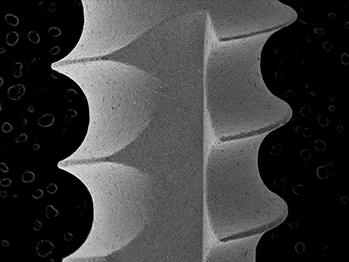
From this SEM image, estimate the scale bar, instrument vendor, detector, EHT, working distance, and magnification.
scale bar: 1000000 nm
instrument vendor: other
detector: SE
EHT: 15 kV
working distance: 41.6 mm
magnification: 0.035 K X